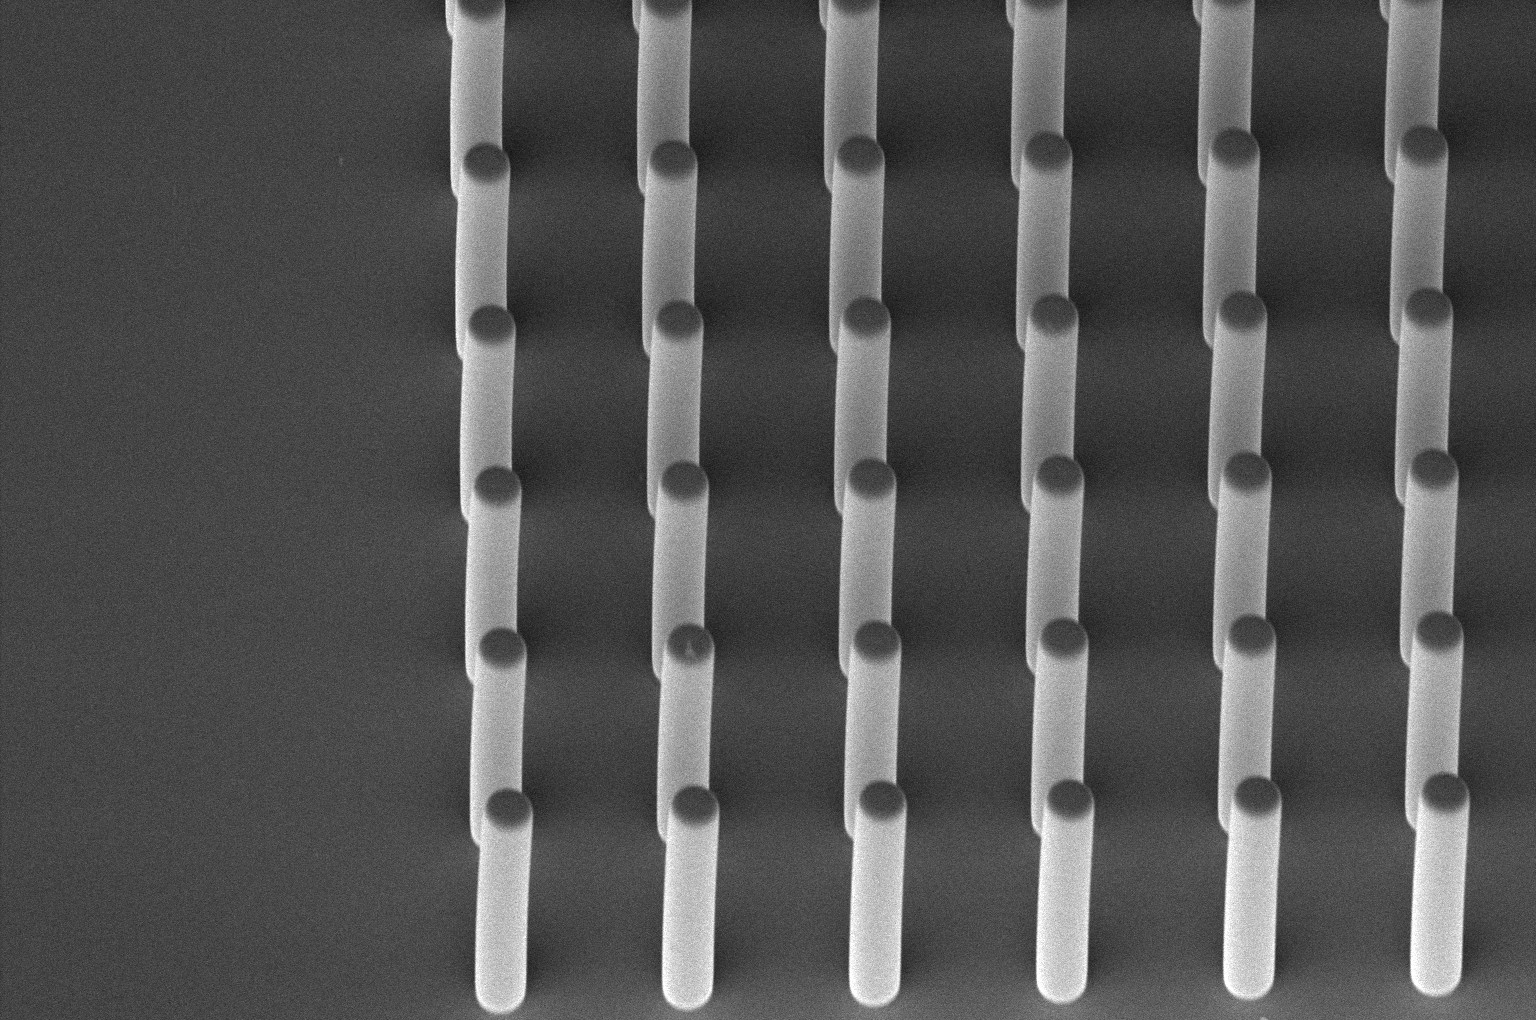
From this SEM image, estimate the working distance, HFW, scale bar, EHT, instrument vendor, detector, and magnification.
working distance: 3.9 mm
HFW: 207 µm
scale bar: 50000 nm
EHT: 2 kV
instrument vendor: FEI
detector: TLD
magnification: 1 K X